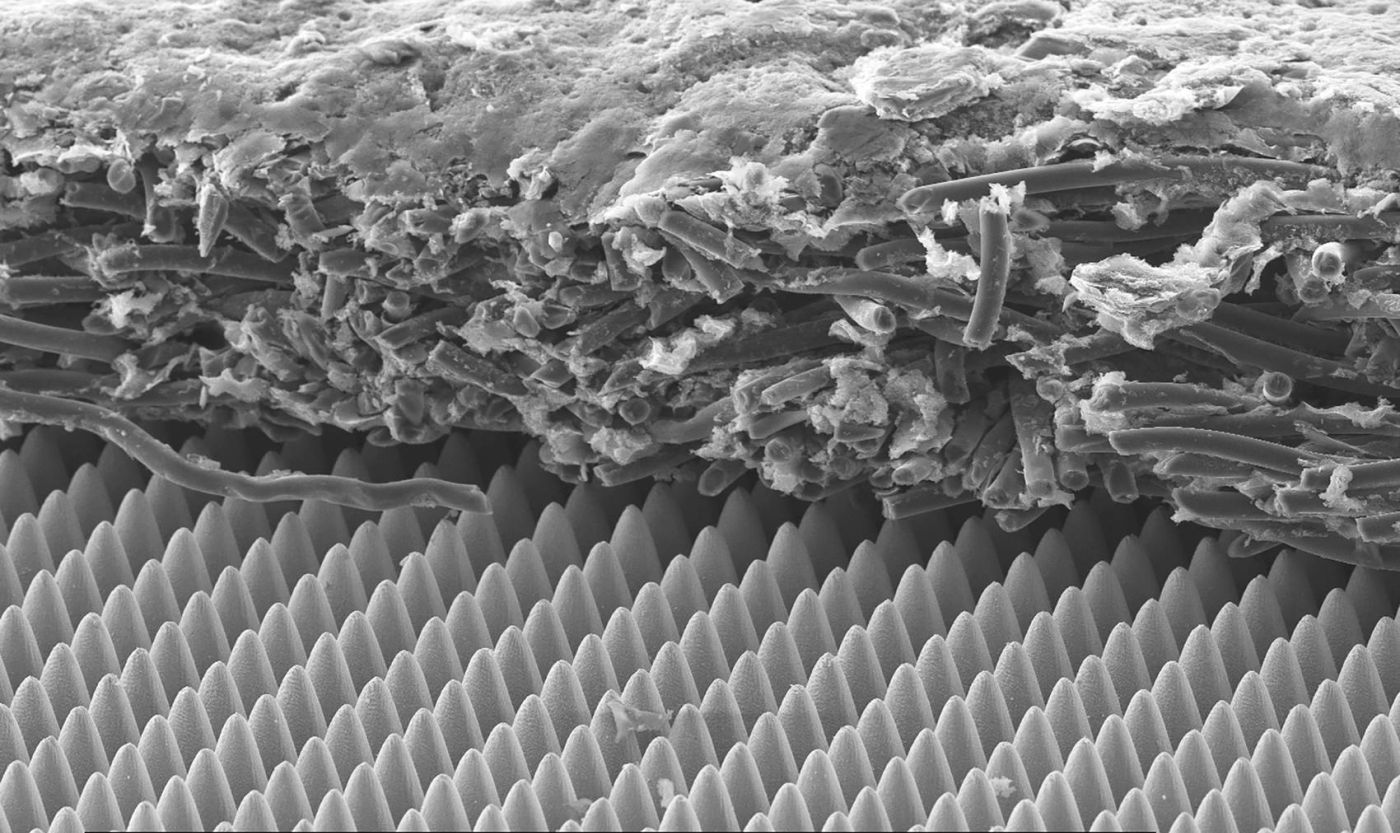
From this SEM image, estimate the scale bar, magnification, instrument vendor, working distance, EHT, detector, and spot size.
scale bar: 100000 nm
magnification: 0.25 K X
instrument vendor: FEI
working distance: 10.7 mm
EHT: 15 kV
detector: SE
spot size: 3